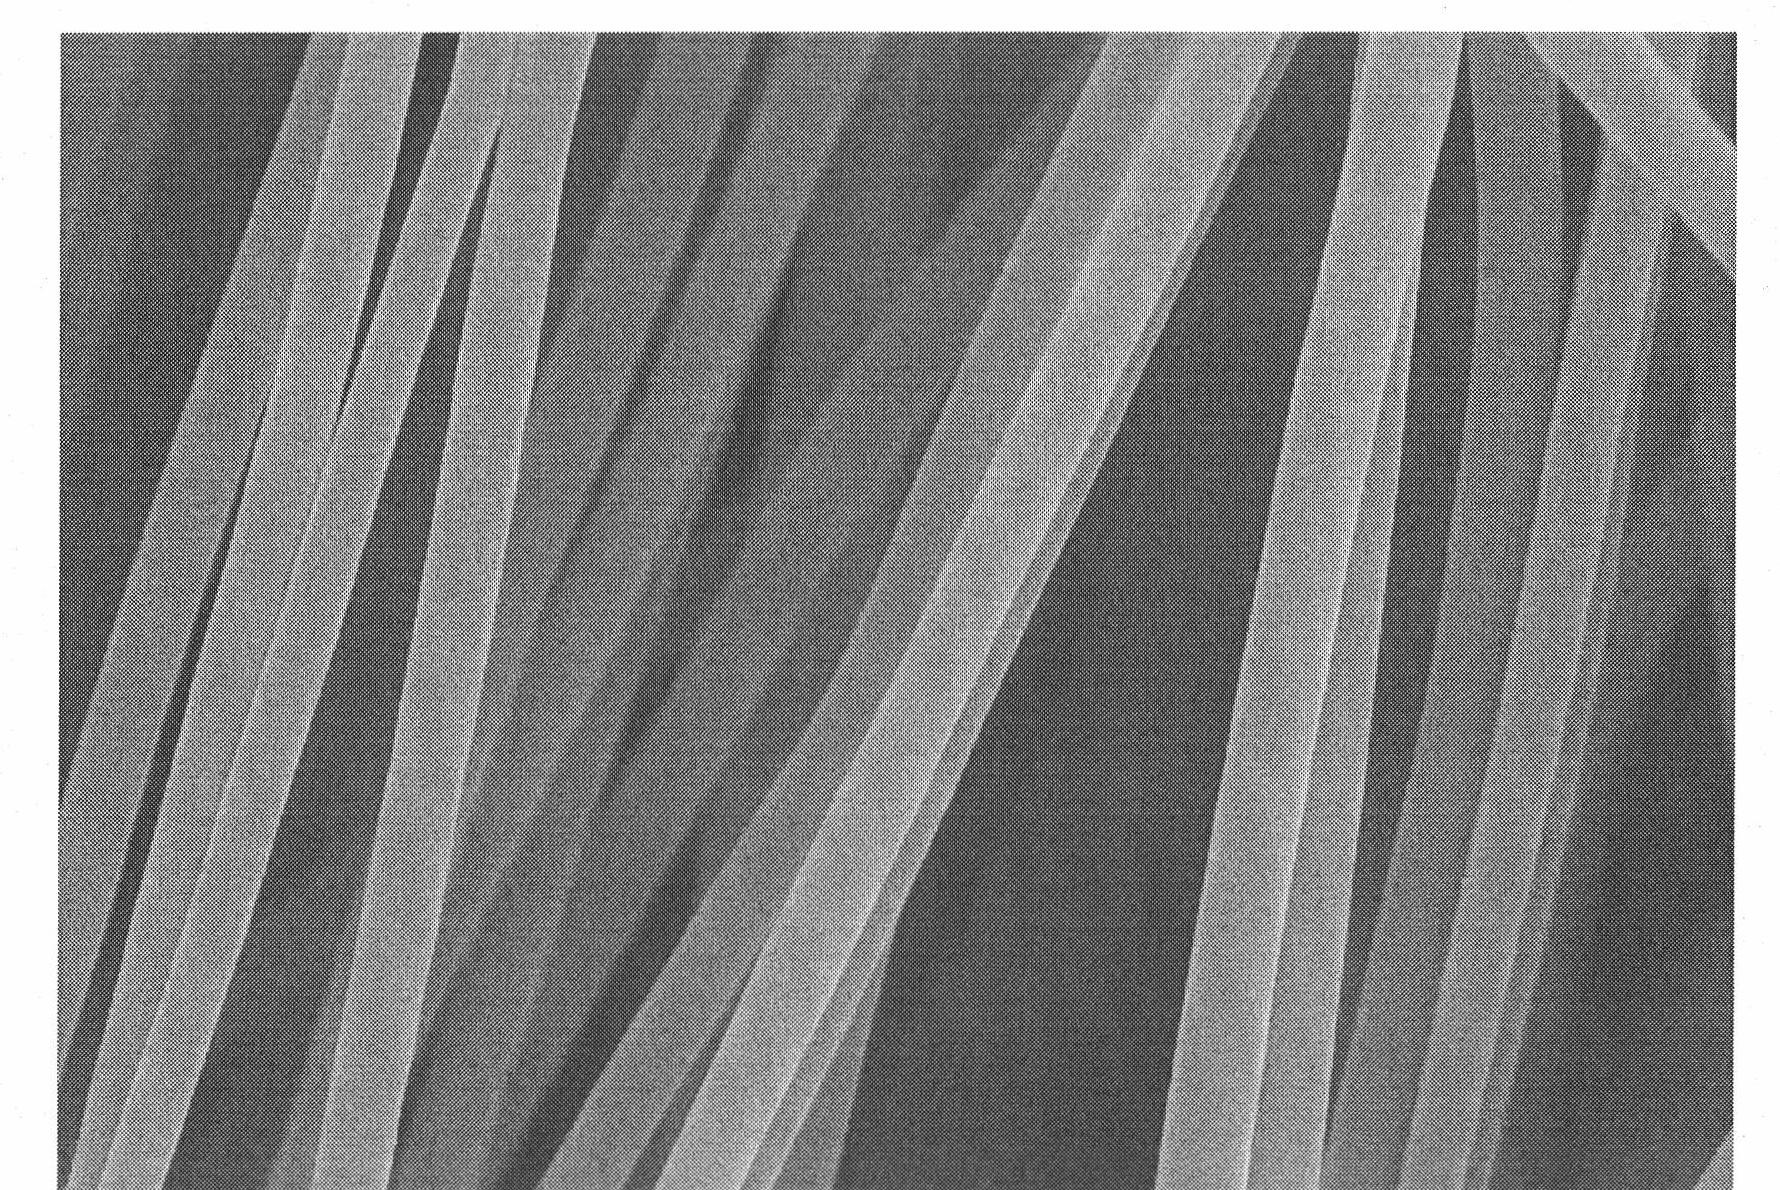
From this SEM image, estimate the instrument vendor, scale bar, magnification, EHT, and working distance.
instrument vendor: Hitachi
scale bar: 2000 nm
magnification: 20 K X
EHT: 15 kV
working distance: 12.2 mm